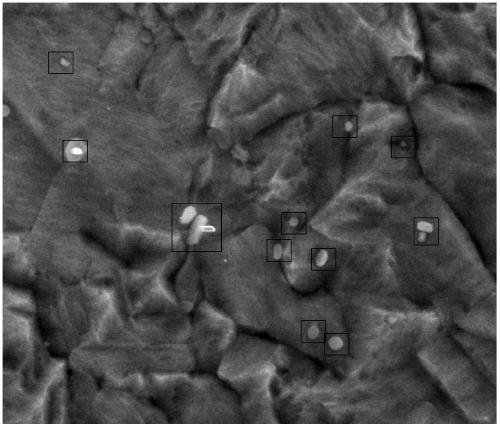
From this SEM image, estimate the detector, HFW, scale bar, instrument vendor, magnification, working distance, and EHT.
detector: ETD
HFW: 12.7 µm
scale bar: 5000 nm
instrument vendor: FEI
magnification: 10 K X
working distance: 10 mm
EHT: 20 kV